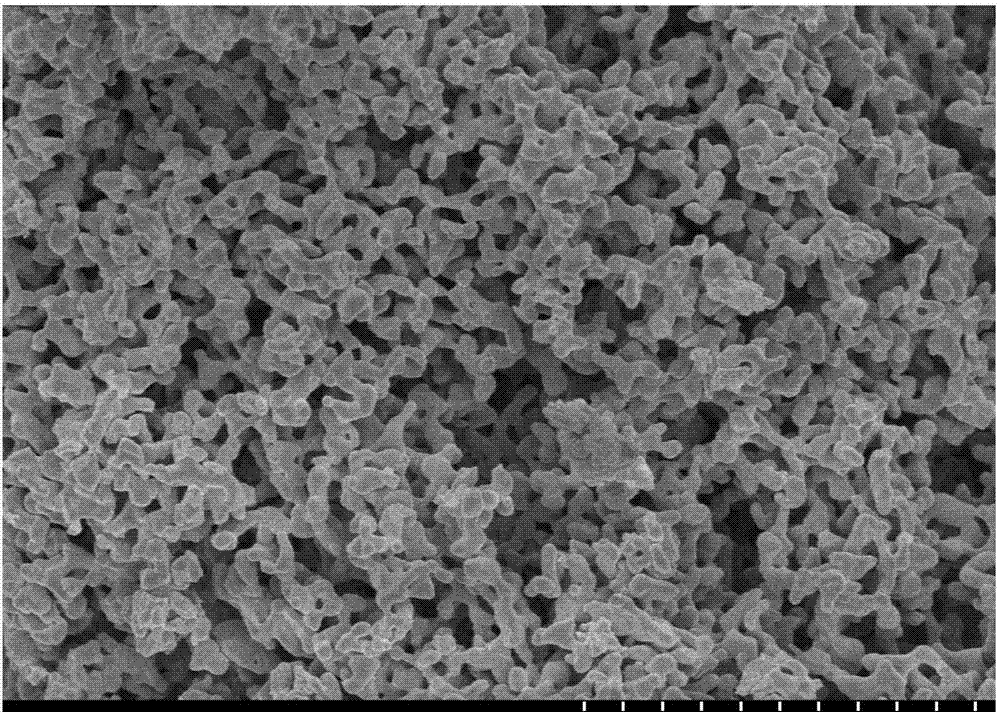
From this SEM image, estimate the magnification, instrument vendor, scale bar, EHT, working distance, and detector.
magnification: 10 K X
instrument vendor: Hitachi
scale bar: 5000 nm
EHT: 3 kV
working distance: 7 mm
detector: SE(M)